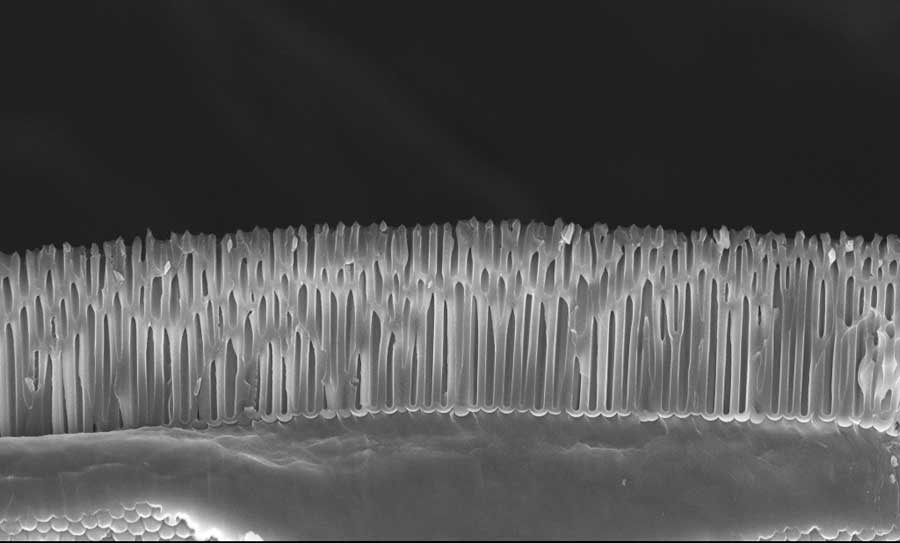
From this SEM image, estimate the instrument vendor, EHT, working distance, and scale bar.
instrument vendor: Zeiss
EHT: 10 kV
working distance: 4 mm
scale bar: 2000 nm